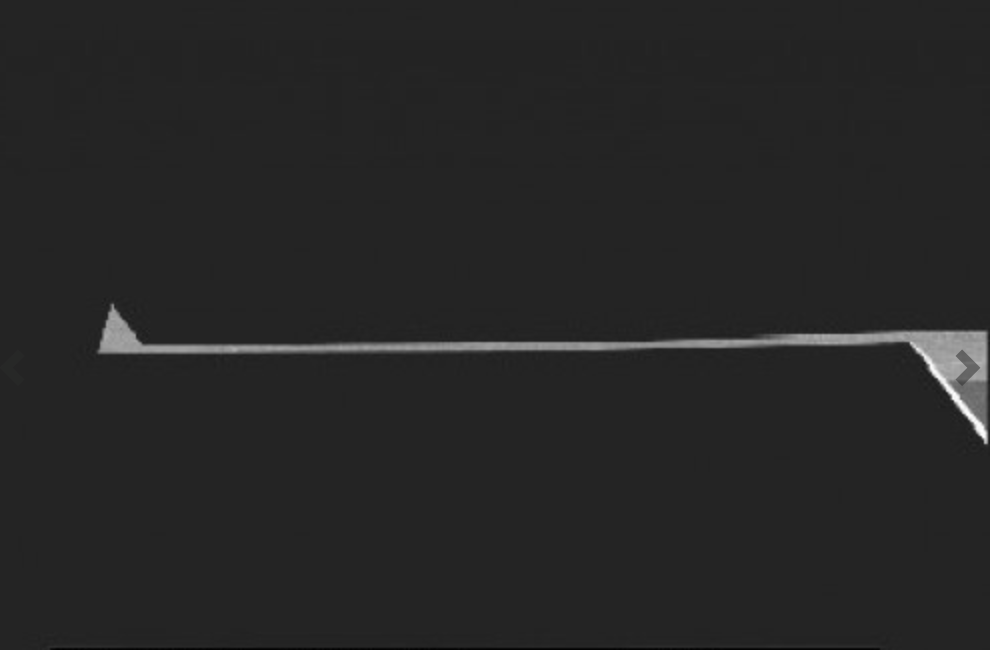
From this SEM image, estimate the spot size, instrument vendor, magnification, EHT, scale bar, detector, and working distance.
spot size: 3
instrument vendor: FEI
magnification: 1 K X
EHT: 5 kV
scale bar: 50000 nm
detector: SE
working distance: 5.2 mm